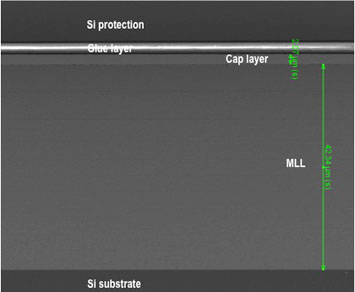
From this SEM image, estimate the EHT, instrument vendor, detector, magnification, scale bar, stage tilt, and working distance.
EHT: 5 kV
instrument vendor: FEI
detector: ETD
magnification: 1.751 K X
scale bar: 30000 nm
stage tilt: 0°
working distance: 4 mm